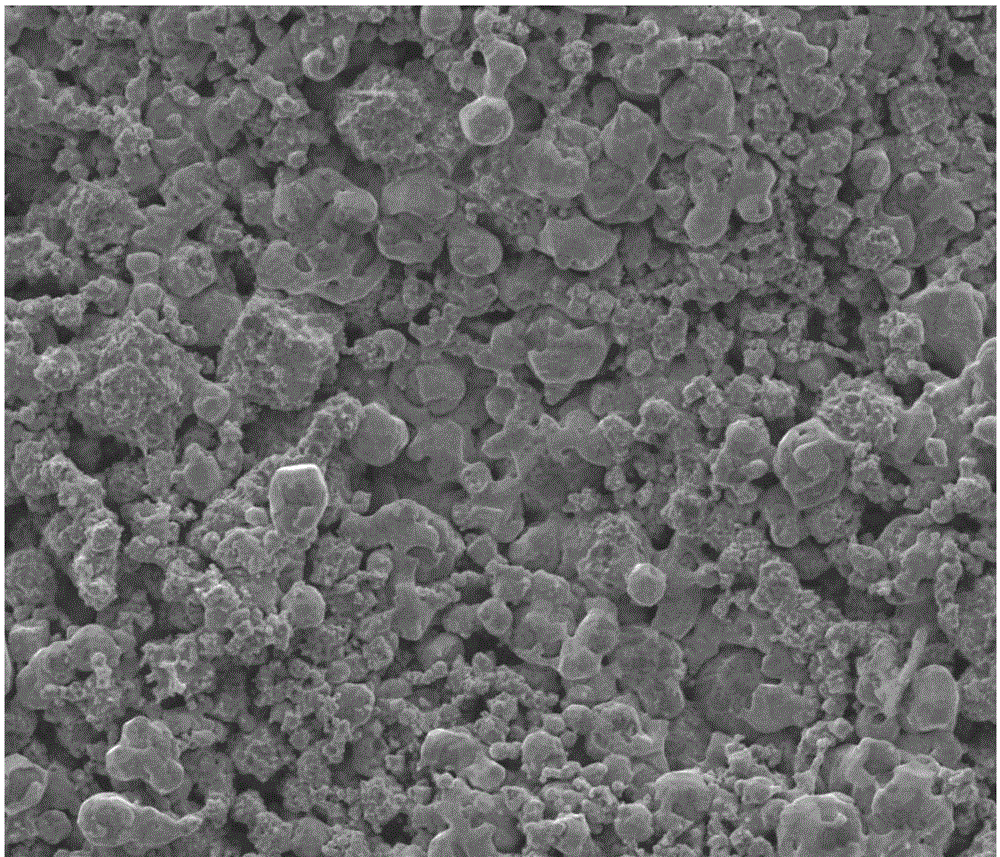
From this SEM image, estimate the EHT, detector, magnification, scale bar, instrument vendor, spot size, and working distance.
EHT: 10 kV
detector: ETD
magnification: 5 K X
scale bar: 10000 nm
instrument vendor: FEI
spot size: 3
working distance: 12.1 mm